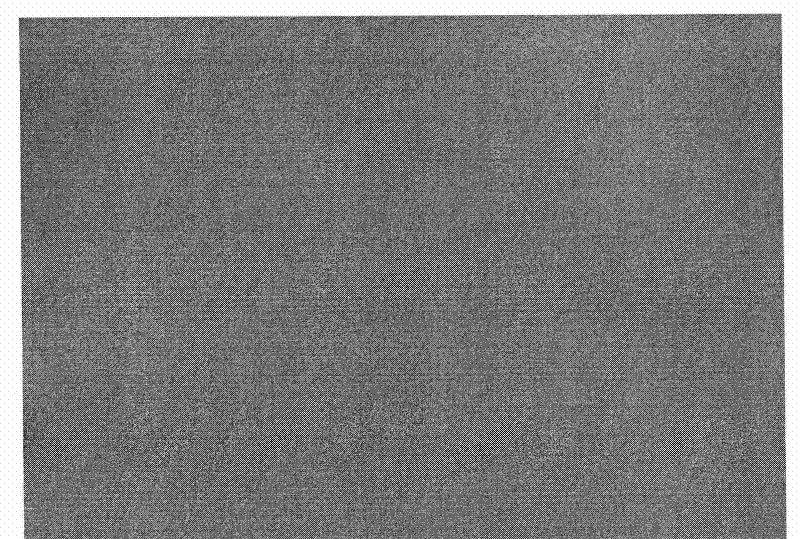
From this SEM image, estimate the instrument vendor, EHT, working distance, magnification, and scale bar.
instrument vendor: Hitachi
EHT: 20 kV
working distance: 12.6 mm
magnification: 10 K X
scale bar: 5000 nm